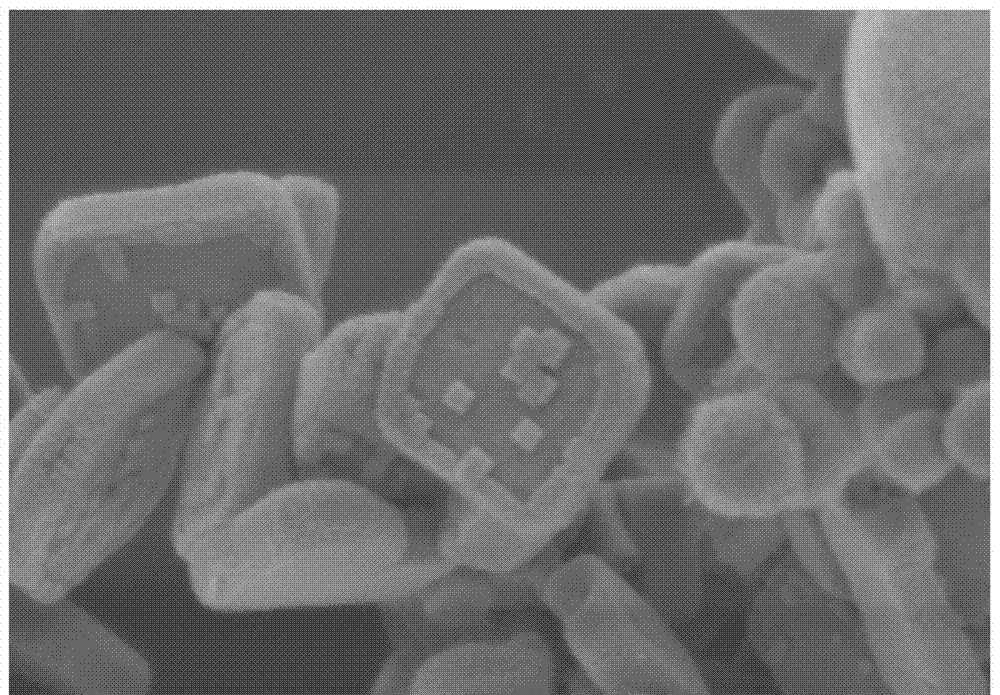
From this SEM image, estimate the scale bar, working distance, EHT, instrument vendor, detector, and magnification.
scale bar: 1000 nm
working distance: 9.1 mm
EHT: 3 kV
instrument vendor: Hitachi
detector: SE(M)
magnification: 50 K X